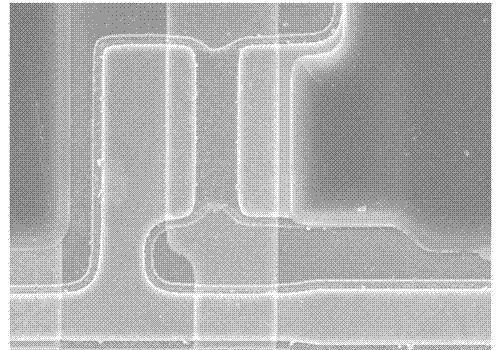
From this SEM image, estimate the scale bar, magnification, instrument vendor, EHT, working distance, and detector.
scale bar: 20000 nm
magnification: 2 K X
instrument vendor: Hitachi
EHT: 15 kV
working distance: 11.4 mm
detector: SE(U)